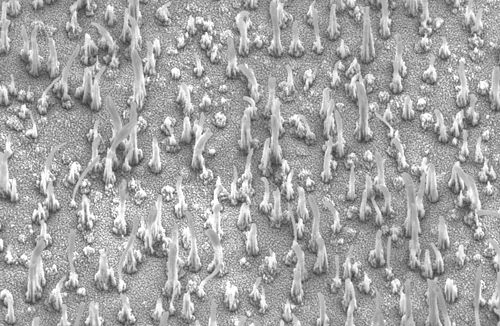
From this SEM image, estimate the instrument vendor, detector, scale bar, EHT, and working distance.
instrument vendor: Zeiss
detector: SE2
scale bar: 1000 nm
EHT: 2.5 kV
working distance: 11.7 mm